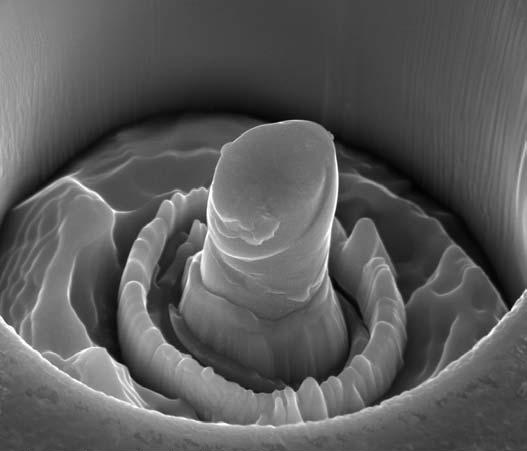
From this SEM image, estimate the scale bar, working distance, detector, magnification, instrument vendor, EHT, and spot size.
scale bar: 5000 nm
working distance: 9.9 mm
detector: ETD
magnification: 10 K X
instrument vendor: FEI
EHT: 20 kV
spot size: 5